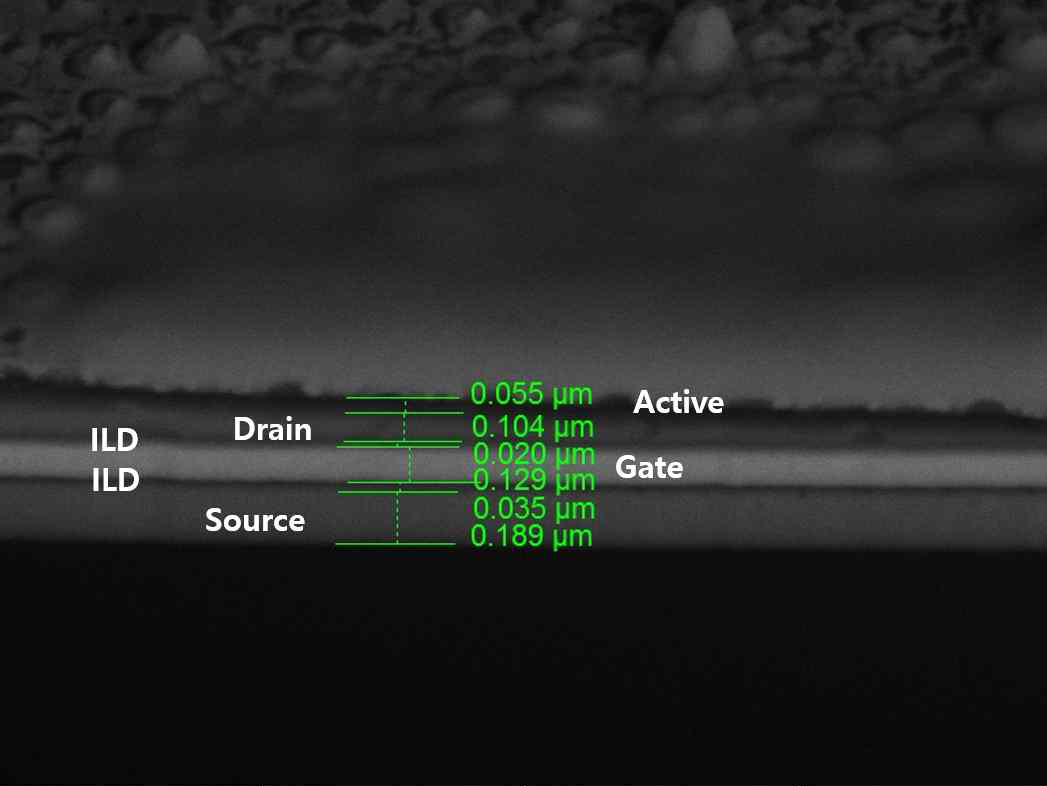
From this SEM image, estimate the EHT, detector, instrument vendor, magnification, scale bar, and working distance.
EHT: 10 kV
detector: BSE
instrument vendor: Tescan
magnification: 50 K X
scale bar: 1000 nm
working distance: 9.93 mm